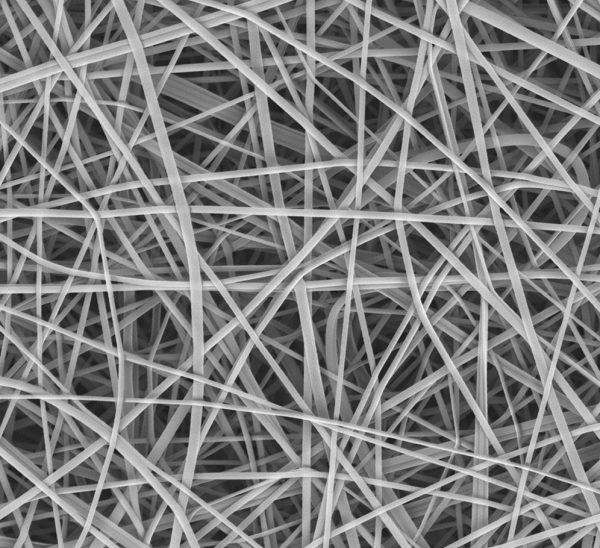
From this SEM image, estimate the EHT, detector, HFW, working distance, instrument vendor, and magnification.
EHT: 10 kV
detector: BSD Full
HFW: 59.8 µm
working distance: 6.721 mm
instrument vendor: other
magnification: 10 K X